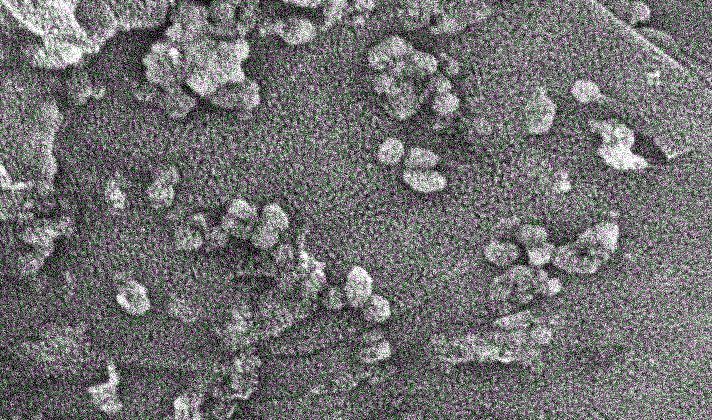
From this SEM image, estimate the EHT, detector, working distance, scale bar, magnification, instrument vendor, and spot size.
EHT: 20 kV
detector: SE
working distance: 4.3 mm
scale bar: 1000 nm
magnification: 20 K X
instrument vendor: FEI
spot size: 2